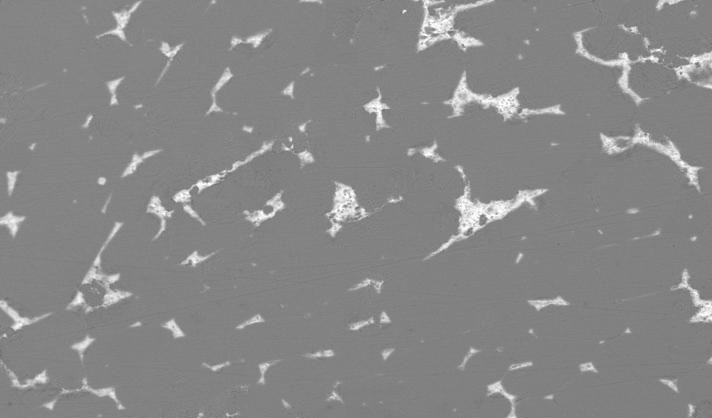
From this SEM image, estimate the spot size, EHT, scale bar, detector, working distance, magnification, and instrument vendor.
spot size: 5.9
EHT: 20 kV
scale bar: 50000 nm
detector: BSE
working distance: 11.7 mm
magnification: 0.5 K X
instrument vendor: FEI